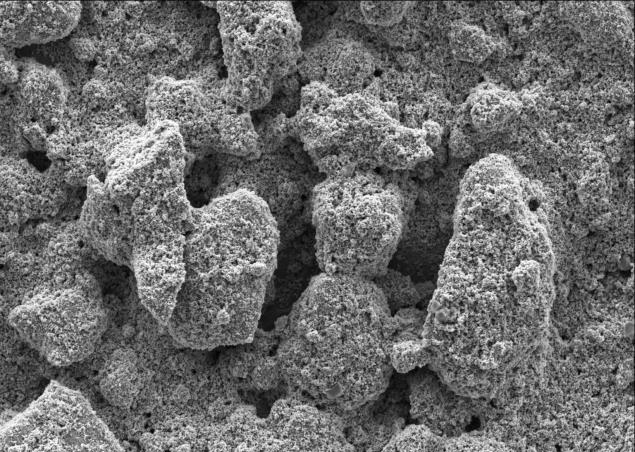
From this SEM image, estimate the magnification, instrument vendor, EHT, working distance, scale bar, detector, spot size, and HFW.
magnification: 6 K X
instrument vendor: FEI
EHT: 5 kV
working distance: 9.9 mm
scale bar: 20000 nm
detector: ETD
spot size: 3.5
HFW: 69.1 µm